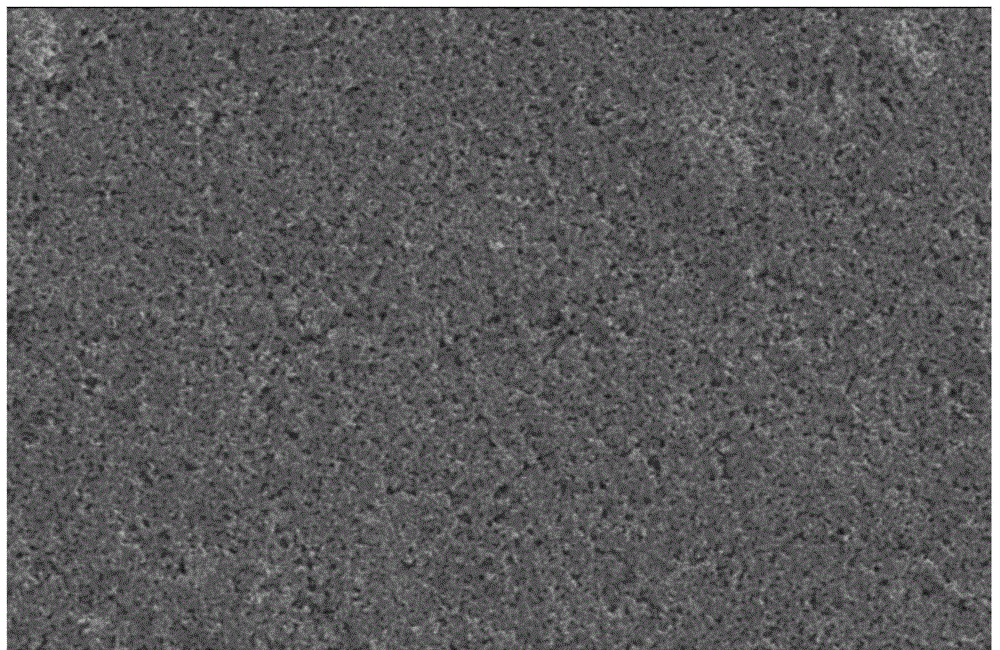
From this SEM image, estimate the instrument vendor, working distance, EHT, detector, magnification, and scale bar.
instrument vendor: Hitachi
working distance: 4.6 mm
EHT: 20 kV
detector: SE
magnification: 1 K X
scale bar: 50000 nm